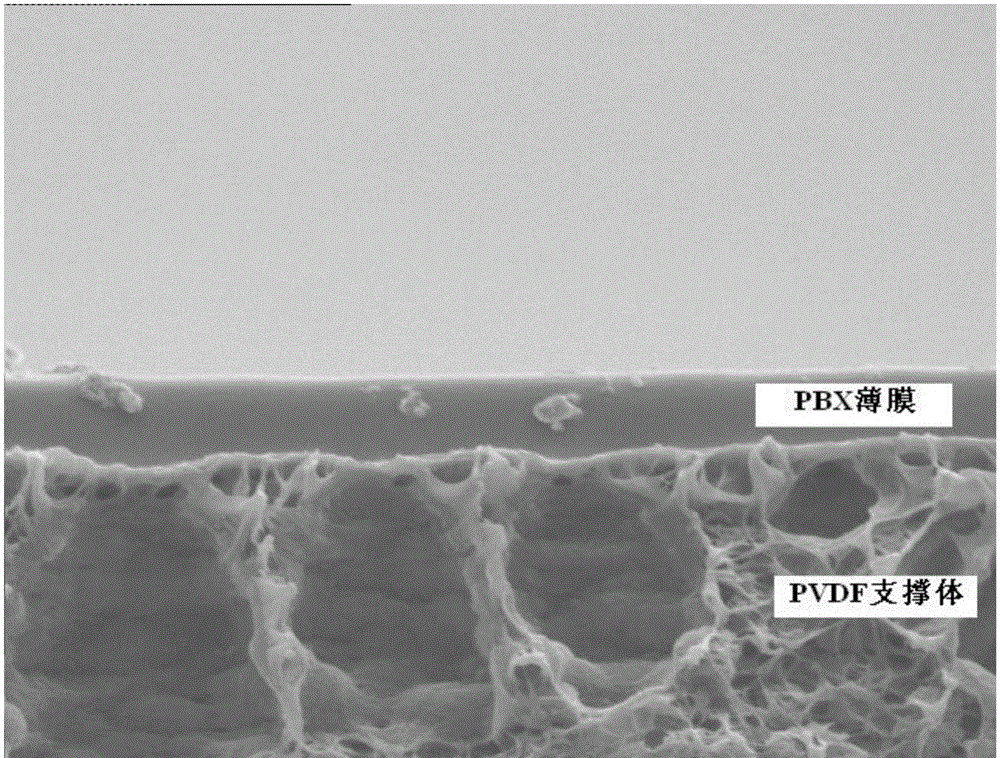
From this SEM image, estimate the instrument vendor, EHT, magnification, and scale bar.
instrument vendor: Hitachi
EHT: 5 kV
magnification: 10 K X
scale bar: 2000 nm